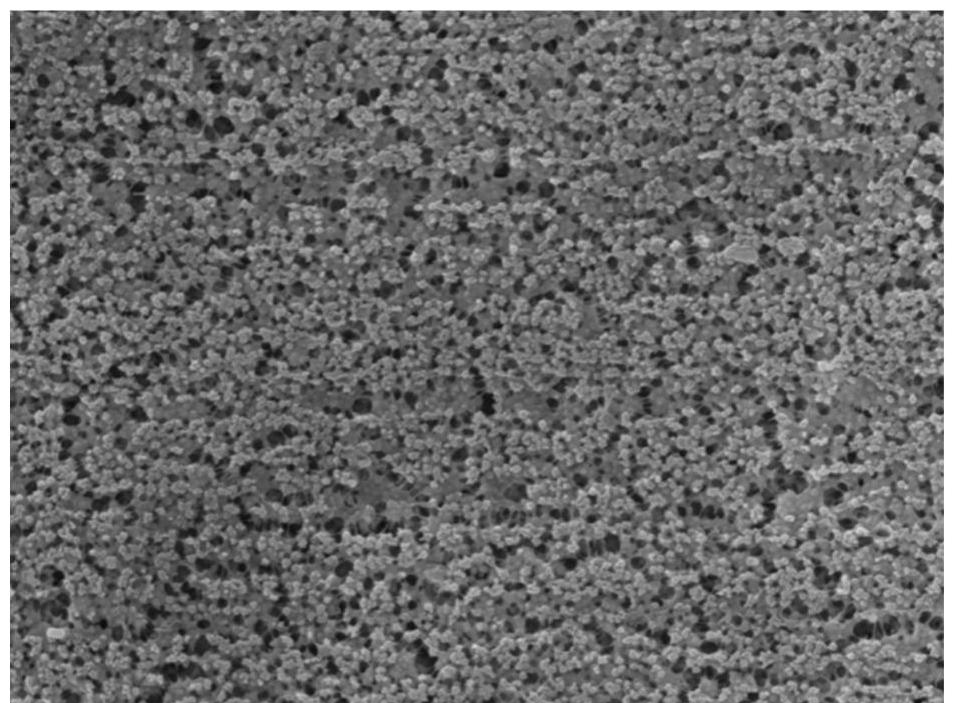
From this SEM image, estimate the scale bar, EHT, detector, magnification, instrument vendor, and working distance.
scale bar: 5000 nm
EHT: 15 kV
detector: SED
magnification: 3 K X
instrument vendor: JEOL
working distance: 10.8 mm